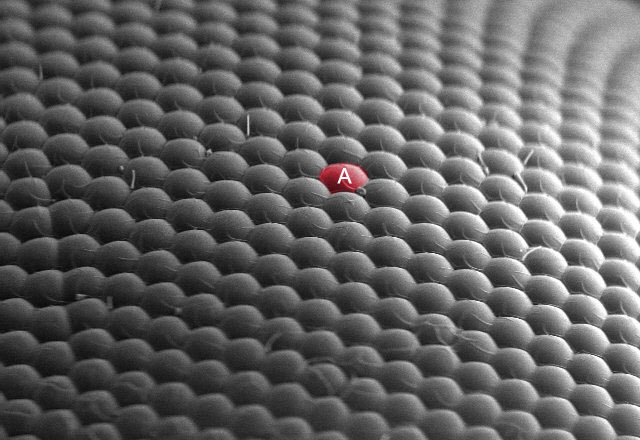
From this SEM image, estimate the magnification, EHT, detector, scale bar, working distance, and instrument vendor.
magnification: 0.457 K X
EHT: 11 kV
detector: SE(M)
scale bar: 100000 nm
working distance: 7.5 mm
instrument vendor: Hitachi